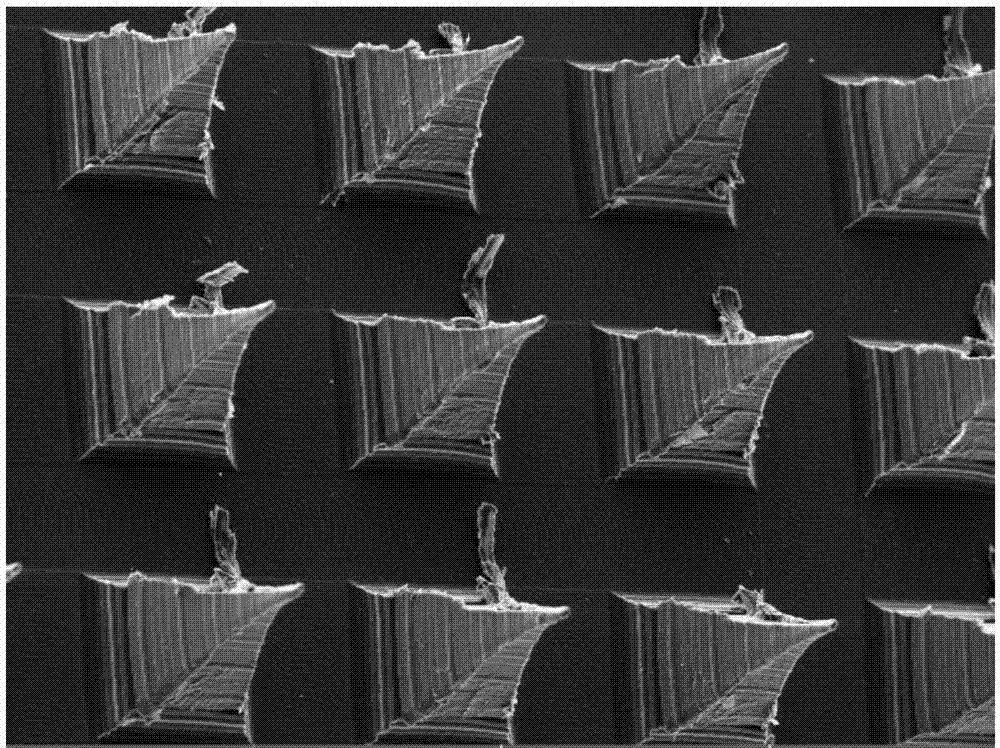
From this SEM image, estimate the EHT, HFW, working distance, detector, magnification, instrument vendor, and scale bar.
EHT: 20 kV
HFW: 1840 µm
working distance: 12.95 mm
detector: SE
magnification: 0.15 K X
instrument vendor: Tescan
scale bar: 500000 nm